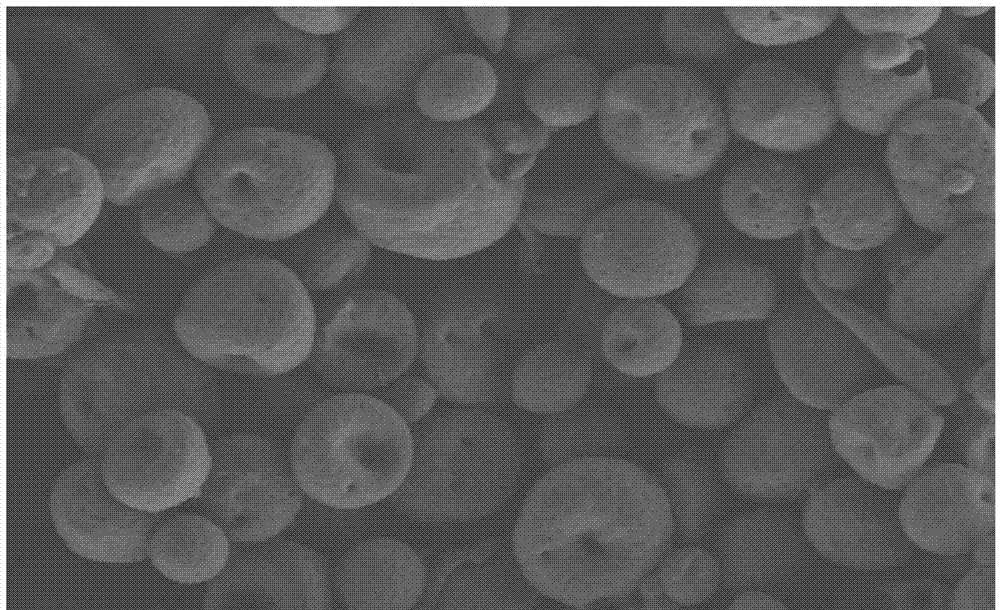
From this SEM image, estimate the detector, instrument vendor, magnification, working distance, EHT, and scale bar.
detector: SE(L)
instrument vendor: Hitachi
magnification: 1 K X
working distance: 8.2 mm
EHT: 1 kV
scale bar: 50000 nm